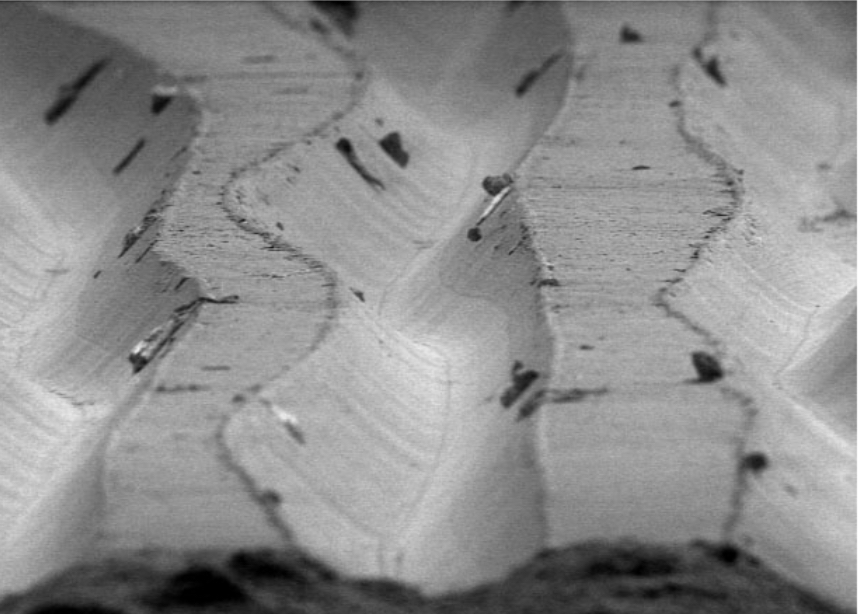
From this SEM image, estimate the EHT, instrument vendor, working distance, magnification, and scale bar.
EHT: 5 kV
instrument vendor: other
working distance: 27 mm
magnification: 0.5 K X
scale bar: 50000 nm